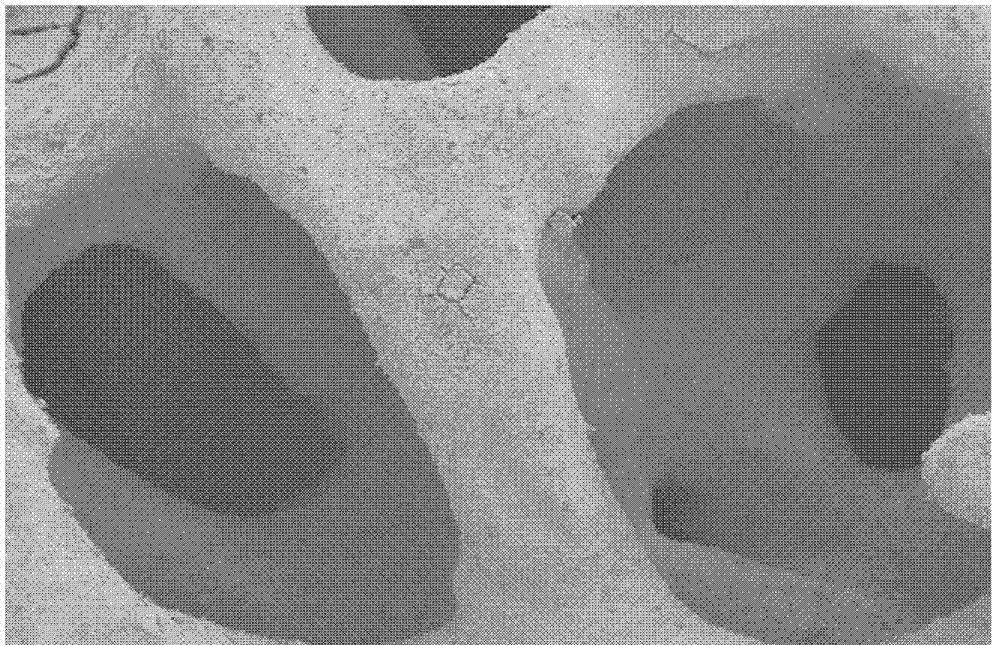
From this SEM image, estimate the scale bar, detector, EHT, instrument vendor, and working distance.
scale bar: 200000 nm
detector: SE2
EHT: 8 kV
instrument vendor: Zeiss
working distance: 14 mm